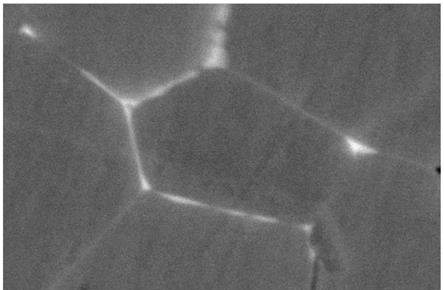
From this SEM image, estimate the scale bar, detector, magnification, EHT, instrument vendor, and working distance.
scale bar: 200 nm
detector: HDBSD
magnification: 20 K X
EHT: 20 kV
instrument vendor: Zeiss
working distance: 8.7 mm